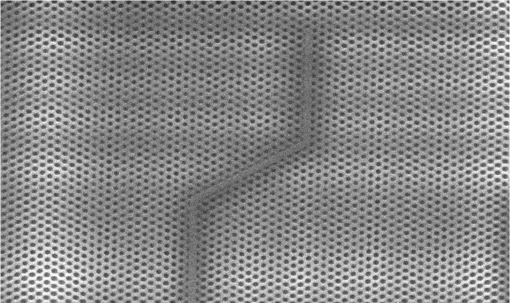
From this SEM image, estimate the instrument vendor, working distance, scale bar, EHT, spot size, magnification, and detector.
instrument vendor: FEI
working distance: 9.6 mm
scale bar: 20000 nm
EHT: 20 kV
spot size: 2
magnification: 1.6 K X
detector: SE